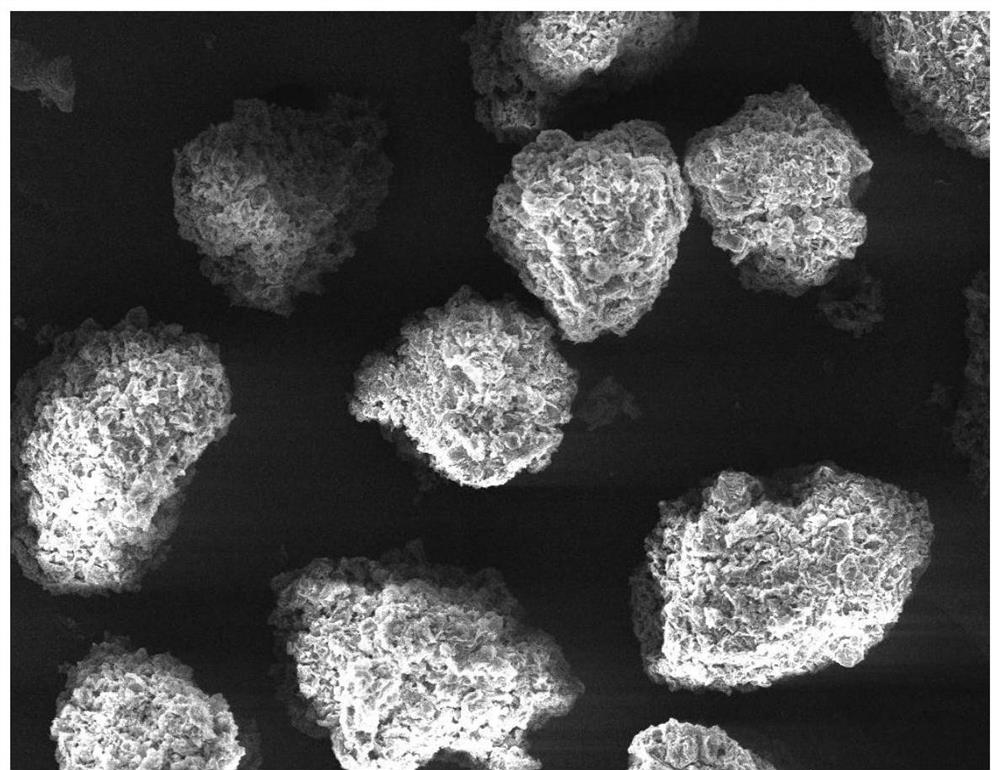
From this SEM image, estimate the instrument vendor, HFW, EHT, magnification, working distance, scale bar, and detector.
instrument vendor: Tescan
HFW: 632 µm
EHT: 7 kV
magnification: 0.4 K X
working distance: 12.23 mm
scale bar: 200000 nm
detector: SE